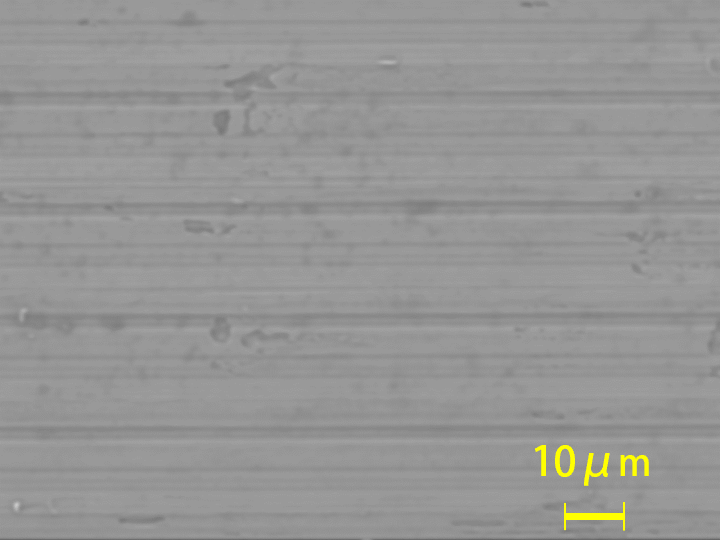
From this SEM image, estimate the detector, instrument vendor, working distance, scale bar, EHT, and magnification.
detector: SED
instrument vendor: JEOL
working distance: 11.9 mm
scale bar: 10000 nm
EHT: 10 kV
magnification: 1 K X